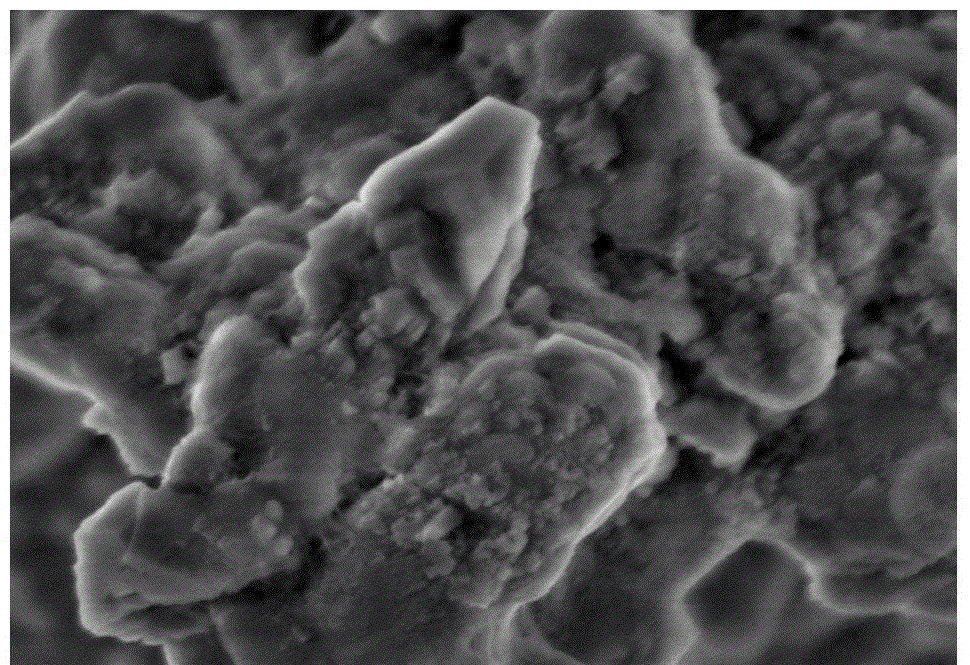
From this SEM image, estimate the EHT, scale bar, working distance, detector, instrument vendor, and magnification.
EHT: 3 kV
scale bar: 1000 nm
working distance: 8.7 mm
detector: SE(M)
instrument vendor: Hitachi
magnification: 50 K X